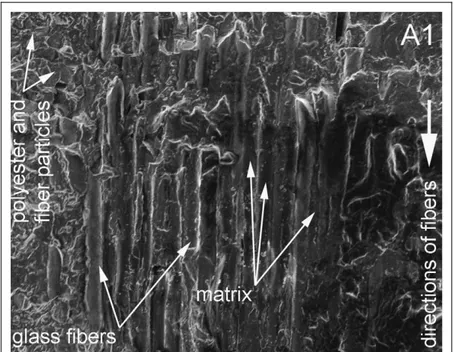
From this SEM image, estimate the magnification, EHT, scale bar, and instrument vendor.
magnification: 0.34 K X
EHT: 10 kV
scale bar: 50000 nm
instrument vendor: JEOL